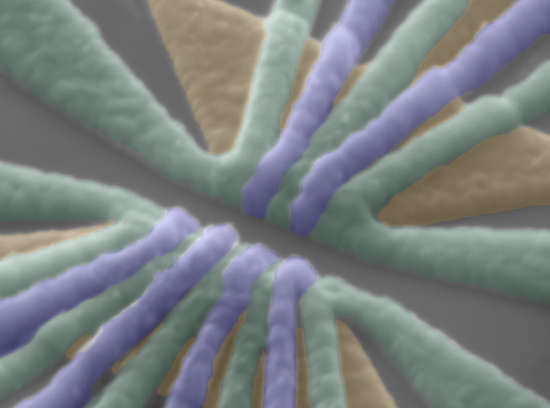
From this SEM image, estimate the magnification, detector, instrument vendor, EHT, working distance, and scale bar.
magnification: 100 K X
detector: LED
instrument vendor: JEOL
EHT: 5 kV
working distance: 8.2 mm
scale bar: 100 nm